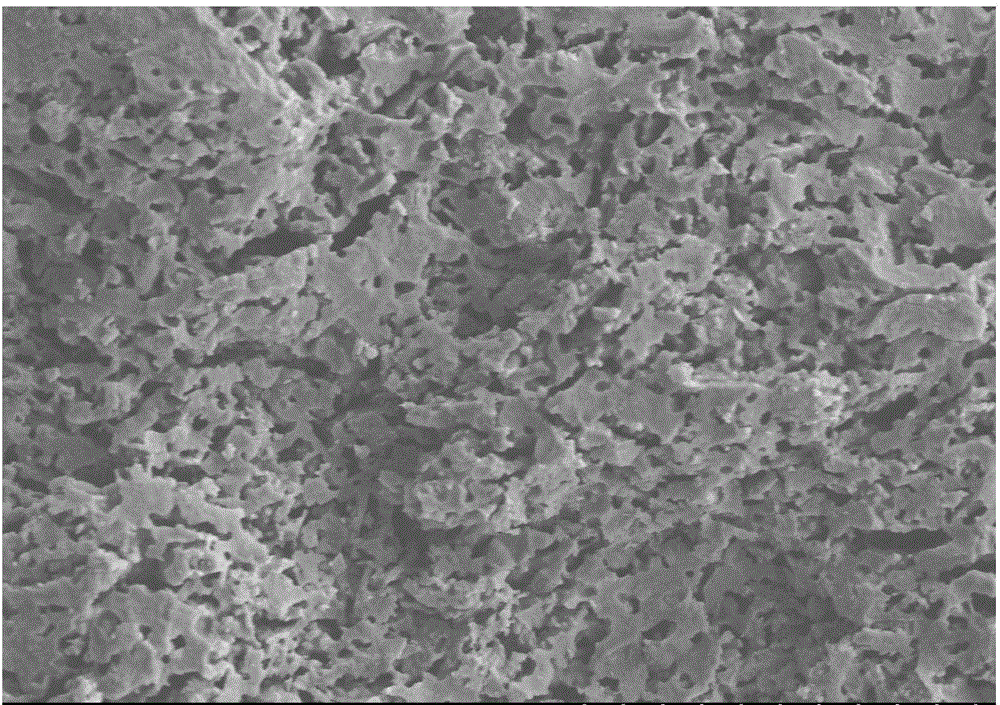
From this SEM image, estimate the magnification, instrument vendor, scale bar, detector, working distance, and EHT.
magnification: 0.5 K X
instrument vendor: Hitachi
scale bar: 100000 nm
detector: SE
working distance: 9 mm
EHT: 15 kV